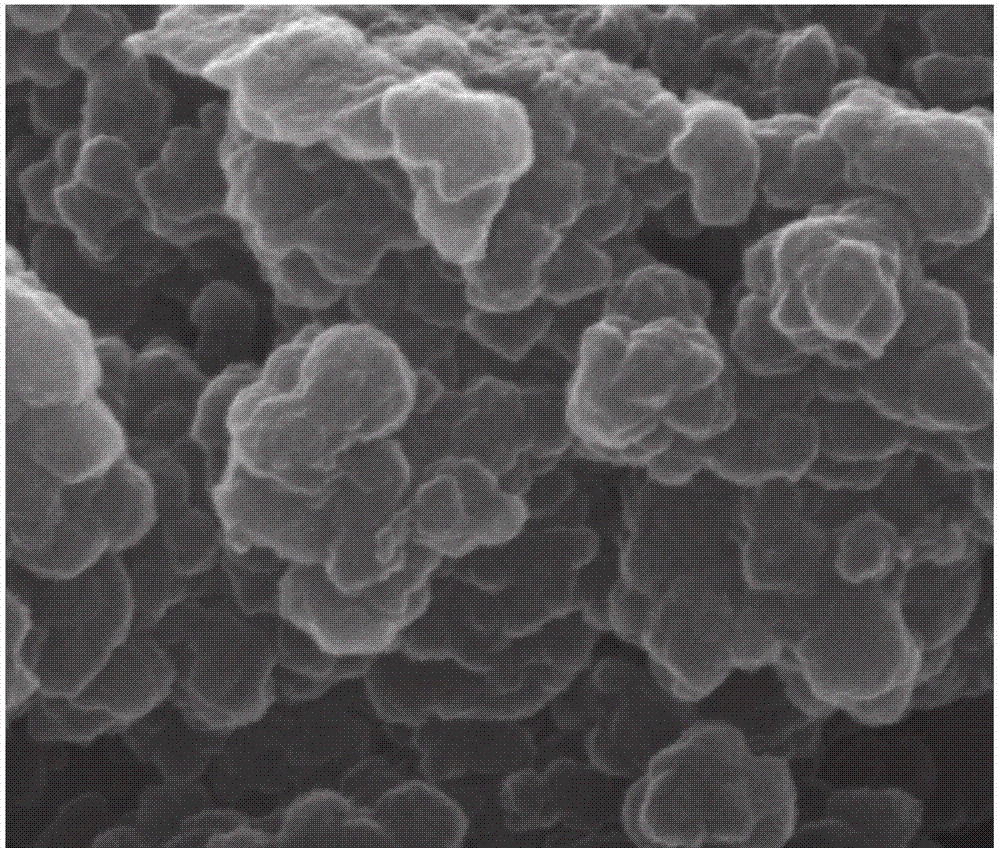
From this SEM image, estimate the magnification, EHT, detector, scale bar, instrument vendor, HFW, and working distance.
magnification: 100 K X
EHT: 20 kV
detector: ETD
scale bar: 1000 nm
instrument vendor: FEI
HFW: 2.98 µm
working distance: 10.5 mm